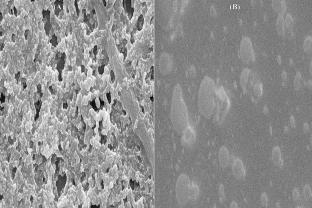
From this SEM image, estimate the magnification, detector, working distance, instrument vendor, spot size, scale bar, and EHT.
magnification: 10 K X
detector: SEI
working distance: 14 mm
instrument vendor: JEOL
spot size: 30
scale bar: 1000 nm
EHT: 15 kV